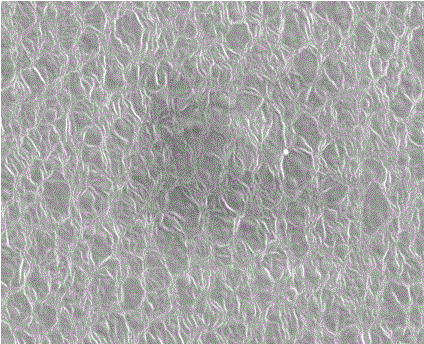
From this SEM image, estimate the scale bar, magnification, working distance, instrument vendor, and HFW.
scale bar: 10000 nm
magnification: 10.053 K X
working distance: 16.4 mm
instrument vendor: FEI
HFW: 32 µm